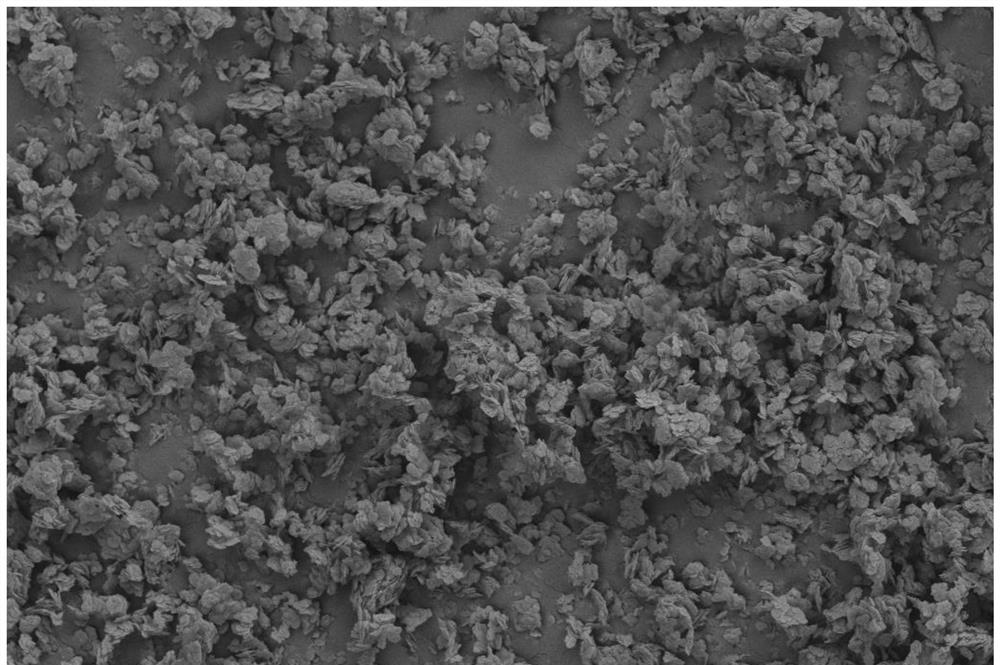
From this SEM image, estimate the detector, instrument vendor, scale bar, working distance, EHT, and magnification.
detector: SE2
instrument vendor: Zeiss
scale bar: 2000 nm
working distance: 9.3 mm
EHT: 5 kV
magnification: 3 K X